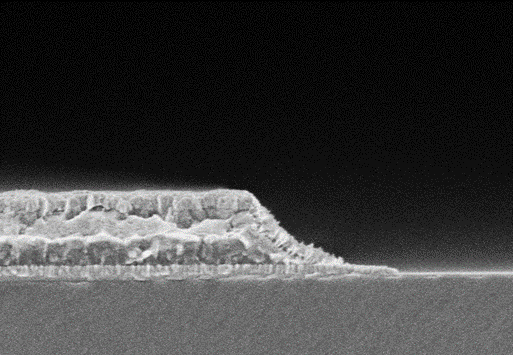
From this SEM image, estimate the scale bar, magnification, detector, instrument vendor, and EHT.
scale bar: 1000 nm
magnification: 50 K X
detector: SE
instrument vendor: Hitachi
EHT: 1.5 kV